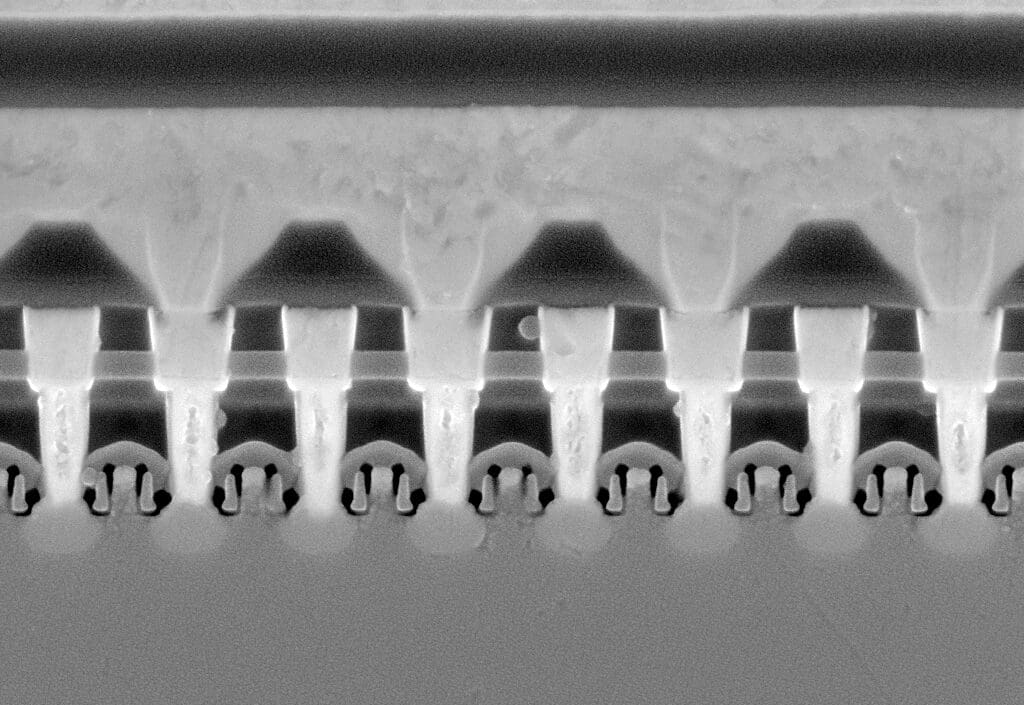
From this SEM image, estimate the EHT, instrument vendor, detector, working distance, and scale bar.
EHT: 10 kV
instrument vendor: Zeiss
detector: InLens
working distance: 5.8 mm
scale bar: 200 nm